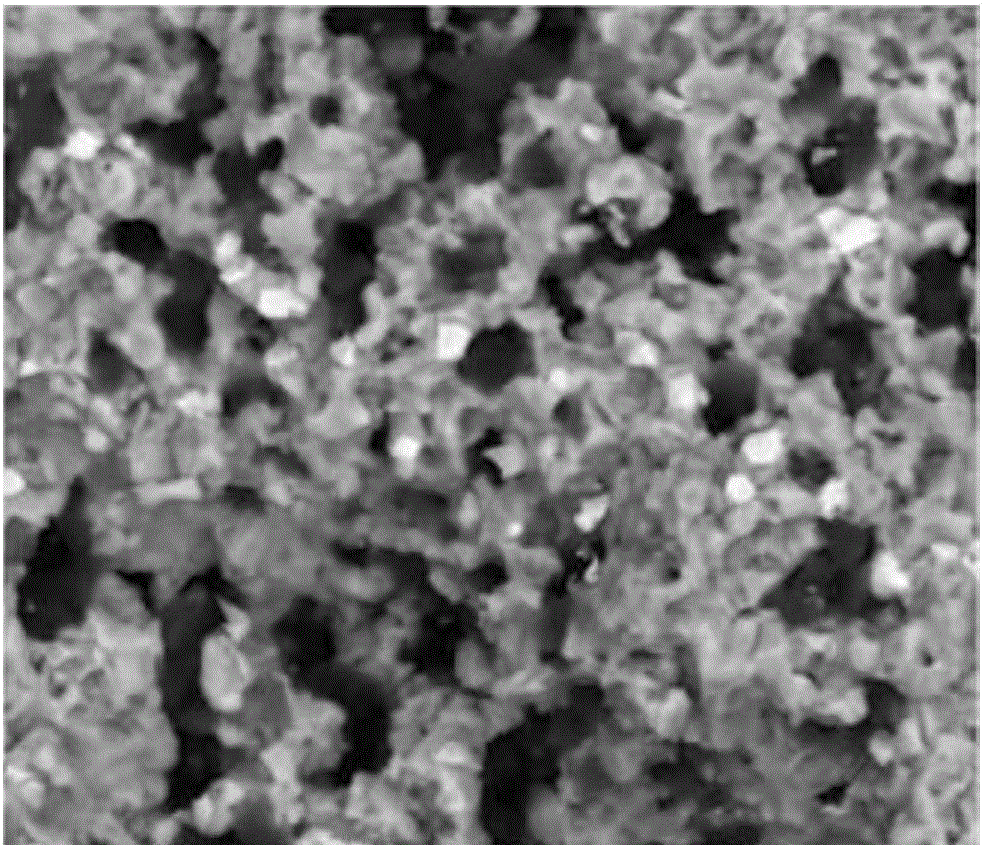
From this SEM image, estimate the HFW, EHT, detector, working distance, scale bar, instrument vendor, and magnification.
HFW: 59.7 µm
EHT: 20 kV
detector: BSED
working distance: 10.3 mm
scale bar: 20000 nm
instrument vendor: FEI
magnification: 5 K X